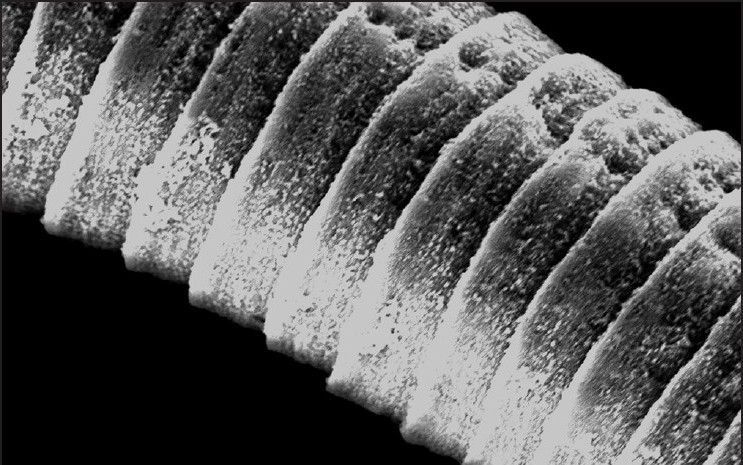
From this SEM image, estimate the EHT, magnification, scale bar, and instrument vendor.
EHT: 20 kV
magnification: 0.6 K X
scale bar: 20000 nm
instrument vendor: JEOL